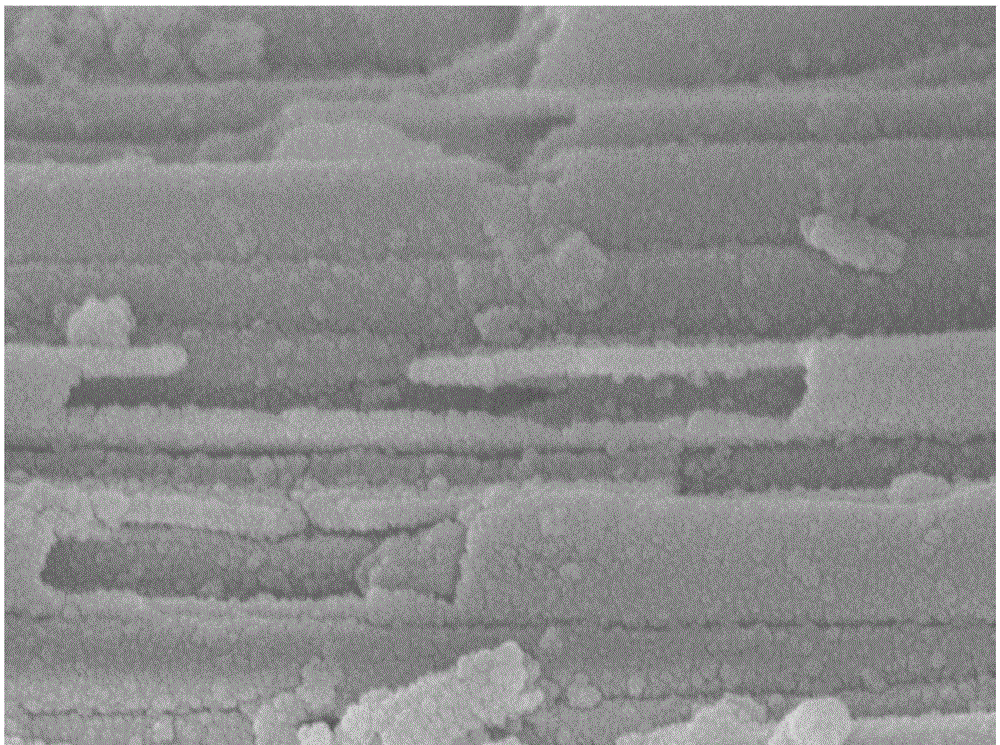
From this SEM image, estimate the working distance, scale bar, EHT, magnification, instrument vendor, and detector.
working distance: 7.9 mm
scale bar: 100 nm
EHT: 8 kV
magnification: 33 K X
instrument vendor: JEOL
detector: SEI